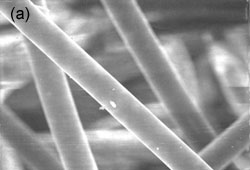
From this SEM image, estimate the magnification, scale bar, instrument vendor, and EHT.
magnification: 0.5 K X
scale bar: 10000 nm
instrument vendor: other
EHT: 20 kV